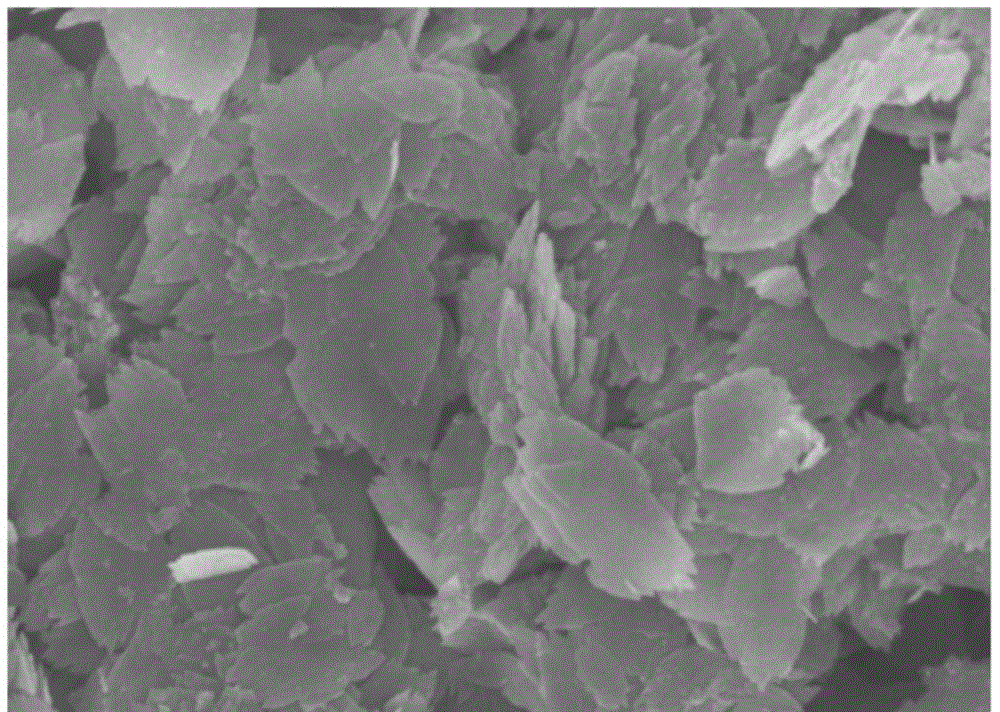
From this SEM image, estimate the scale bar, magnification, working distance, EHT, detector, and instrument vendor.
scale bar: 2000 nm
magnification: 20 K X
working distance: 8.8 mm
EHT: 5 kV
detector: SE(M)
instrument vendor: Hitachi